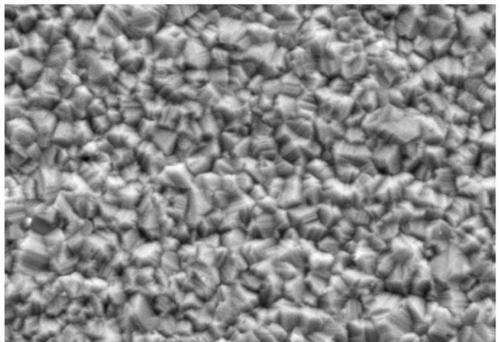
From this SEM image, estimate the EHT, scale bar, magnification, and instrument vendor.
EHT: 20 kV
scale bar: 10000 nm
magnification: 3 K X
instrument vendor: other